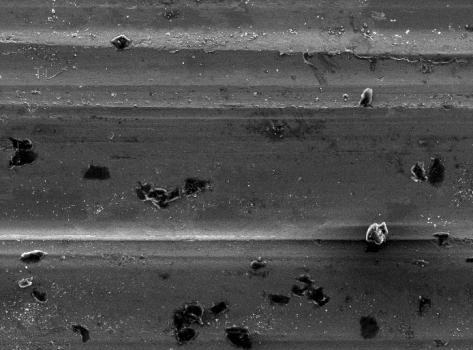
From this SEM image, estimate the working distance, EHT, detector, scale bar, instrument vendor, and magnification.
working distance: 10.1 mm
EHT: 15 kV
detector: SEI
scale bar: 100000 nm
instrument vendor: JEOL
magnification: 0.2 K X